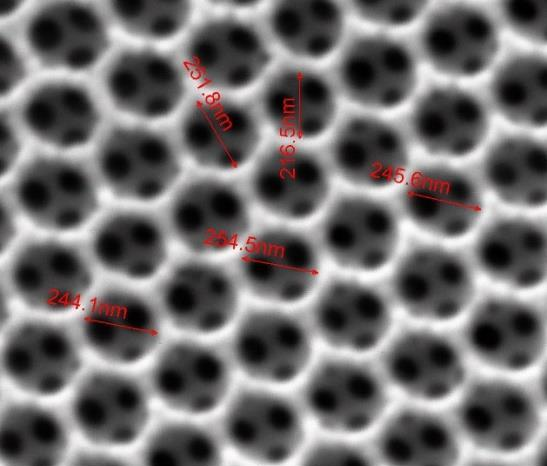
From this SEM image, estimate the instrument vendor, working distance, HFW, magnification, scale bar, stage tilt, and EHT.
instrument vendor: FEI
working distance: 9.8 mm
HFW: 1.8 µm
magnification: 150 K X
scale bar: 500 nm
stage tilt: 0°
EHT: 25 kV